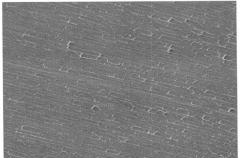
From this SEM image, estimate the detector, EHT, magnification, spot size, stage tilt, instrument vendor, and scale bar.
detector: ETD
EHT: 5 kV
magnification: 4 K X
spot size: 3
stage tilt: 0°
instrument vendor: FEI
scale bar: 10000 nm